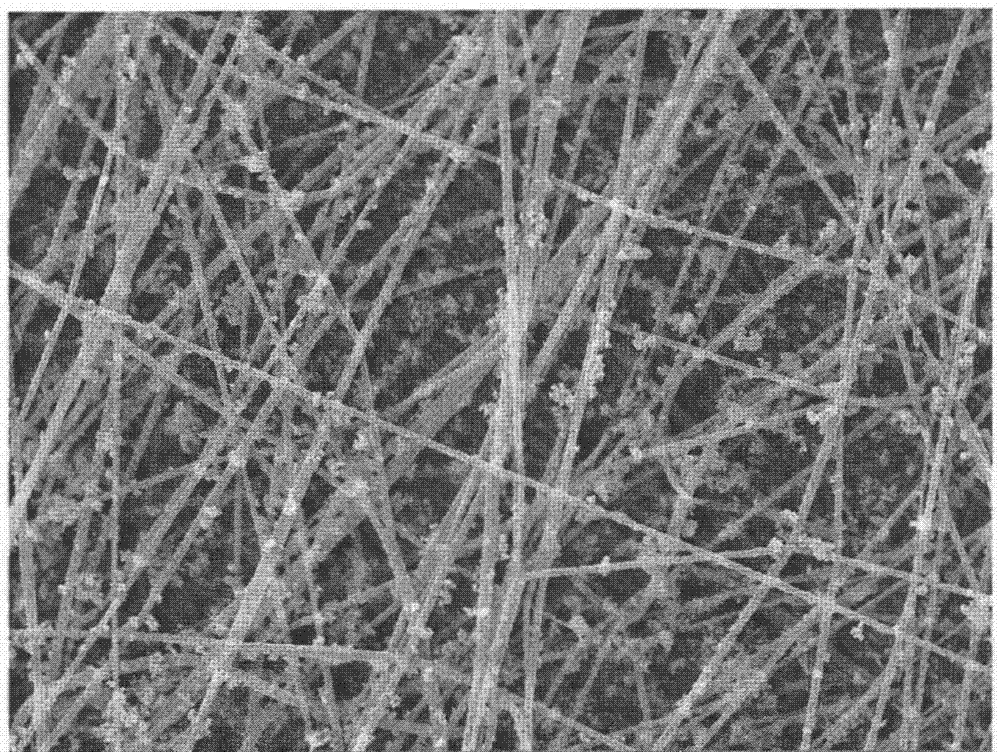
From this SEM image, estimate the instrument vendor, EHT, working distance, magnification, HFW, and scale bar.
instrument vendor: Tescan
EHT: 10 kV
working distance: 7.88 mm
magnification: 10 K X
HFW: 27.7 µm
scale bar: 5000 nm